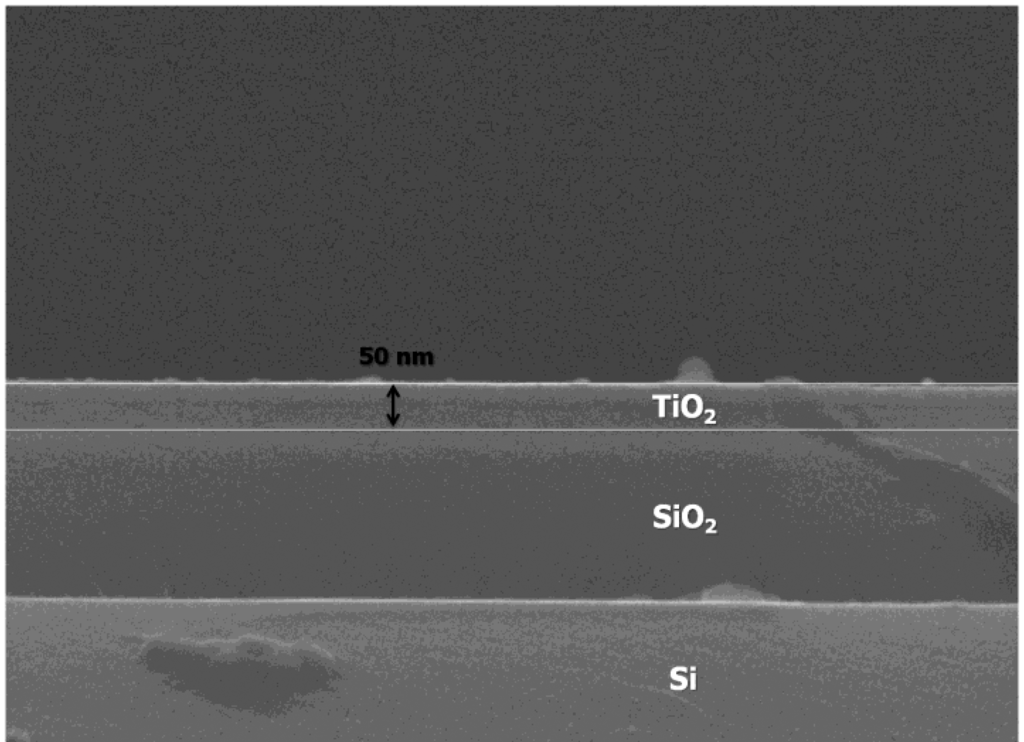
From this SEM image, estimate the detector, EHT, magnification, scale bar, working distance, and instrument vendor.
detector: SEI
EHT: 15 kV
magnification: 100 K X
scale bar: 100 nm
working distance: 9.4 mm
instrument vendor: JEOL